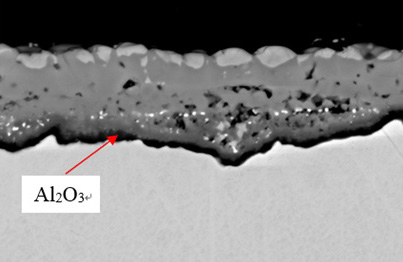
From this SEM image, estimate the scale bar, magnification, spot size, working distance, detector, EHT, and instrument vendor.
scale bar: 5000 nm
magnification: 3 K X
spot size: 60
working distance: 11 mm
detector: BEC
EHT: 20 kV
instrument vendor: JEOL